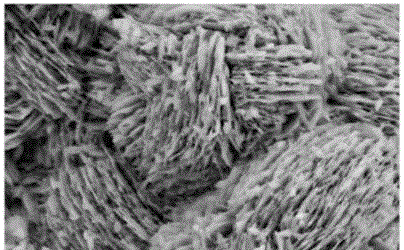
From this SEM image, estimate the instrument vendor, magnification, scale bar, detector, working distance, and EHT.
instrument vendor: Zeiss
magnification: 50 K X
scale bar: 200 nm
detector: SE2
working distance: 7.7 mm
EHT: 5 kV